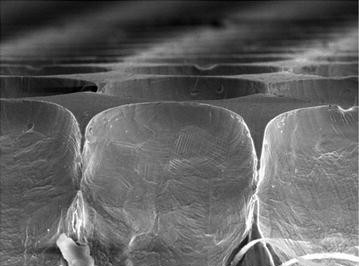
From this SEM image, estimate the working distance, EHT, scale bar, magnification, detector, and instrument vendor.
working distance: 13.8 mm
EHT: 15 kV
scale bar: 50000 nm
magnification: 1 K X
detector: SE(M,LA3)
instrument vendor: Hitachi